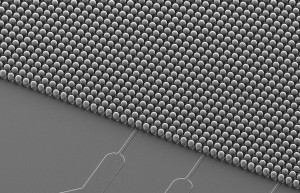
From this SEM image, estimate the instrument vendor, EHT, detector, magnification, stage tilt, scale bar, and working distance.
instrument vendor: Zeiss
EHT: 10 kV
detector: SE2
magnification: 0.4 K X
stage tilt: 45°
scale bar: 10000 nm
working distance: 11 mm